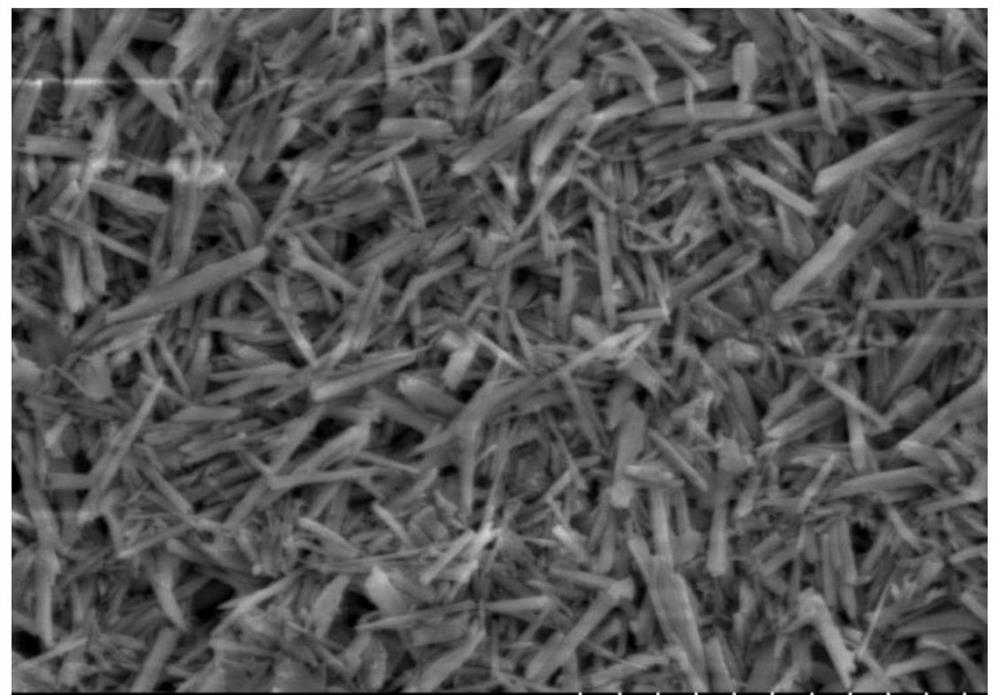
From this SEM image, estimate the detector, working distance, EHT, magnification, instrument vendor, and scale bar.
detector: SE(L)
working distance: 6.5 mm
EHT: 5 kV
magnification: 10 K X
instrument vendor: Hitachi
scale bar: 5000 nm